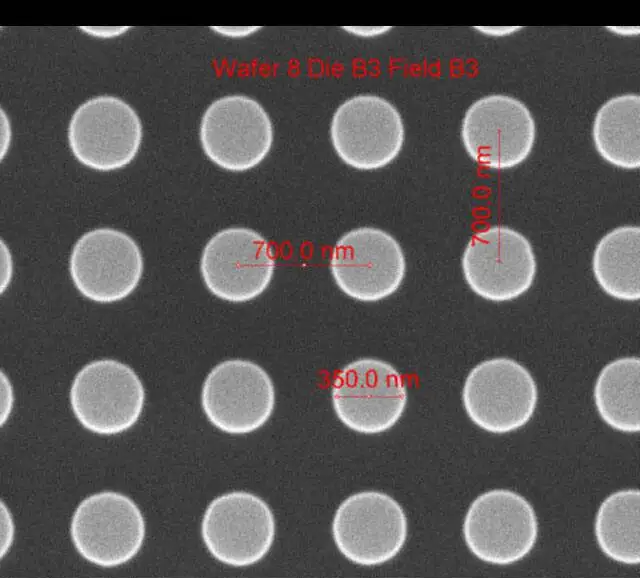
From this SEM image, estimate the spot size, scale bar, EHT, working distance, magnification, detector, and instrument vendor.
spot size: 1.5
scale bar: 1000 nm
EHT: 10 kV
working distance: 10.3 mm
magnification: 75 K X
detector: ETD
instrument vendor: FEI